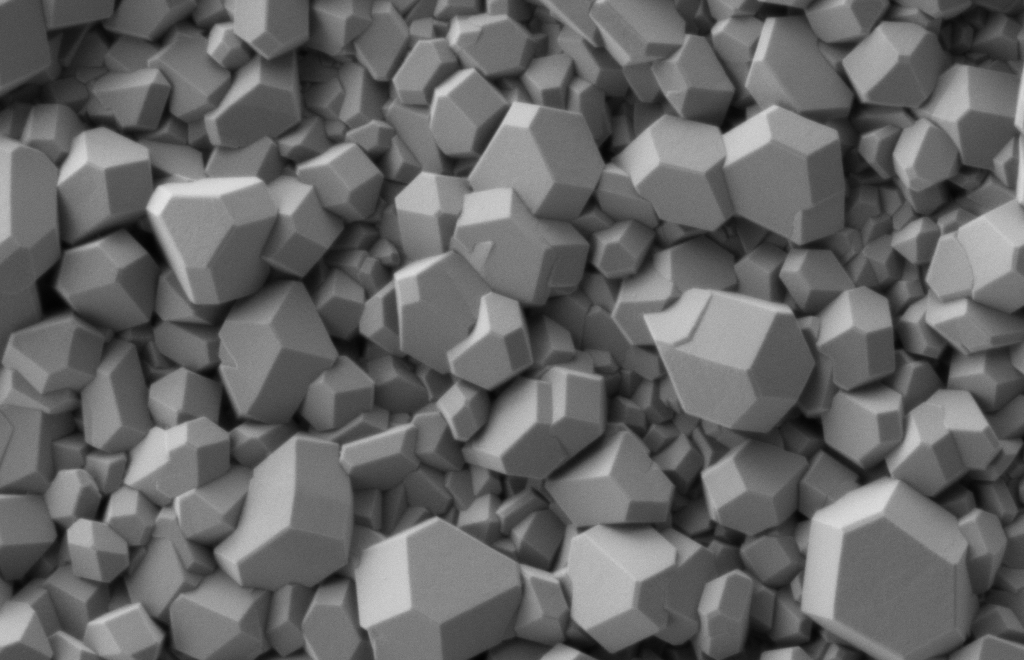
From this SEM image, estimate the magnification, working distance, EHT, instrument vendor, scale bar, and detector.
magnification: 20 K X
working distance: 4.3 mm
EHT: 1 kV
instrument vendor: Zeiss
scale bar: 200 nm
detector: SE2